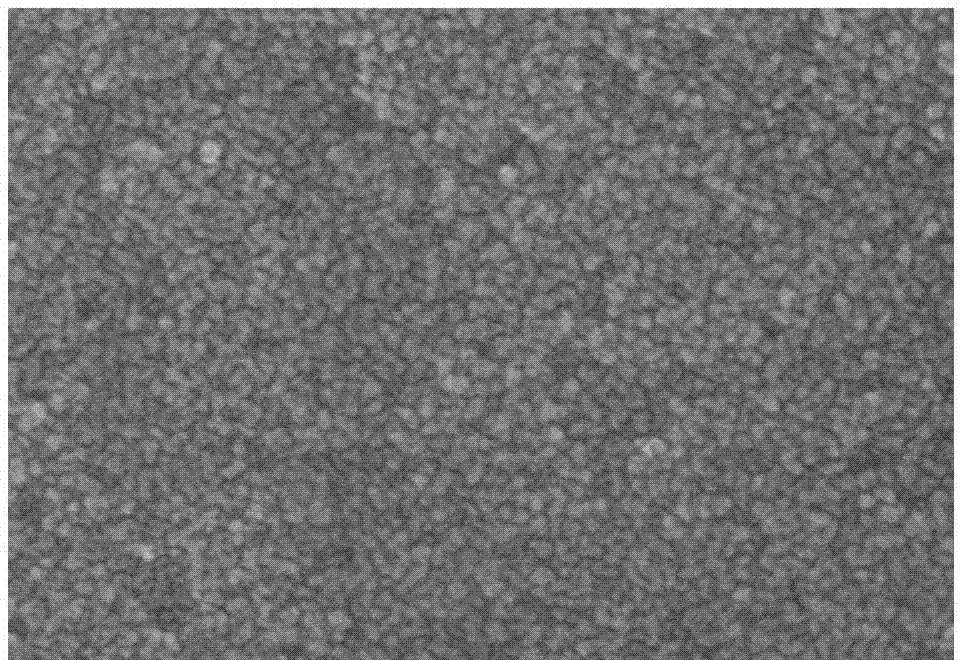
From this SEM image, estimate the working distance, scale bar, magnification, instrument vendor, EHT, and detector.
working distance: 12.9 mm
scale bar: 500 nm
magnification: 80 K X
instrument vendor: Hitachi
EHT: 10 kV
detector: SE(TUL)